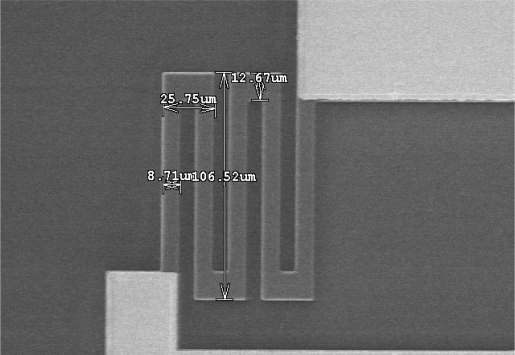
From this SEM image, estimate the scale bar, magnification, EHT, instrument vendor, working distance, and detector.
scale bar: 100000 nm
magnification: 0.5 K X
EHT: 15 kV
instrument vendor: JEOL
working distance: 26.9 mm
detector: SE